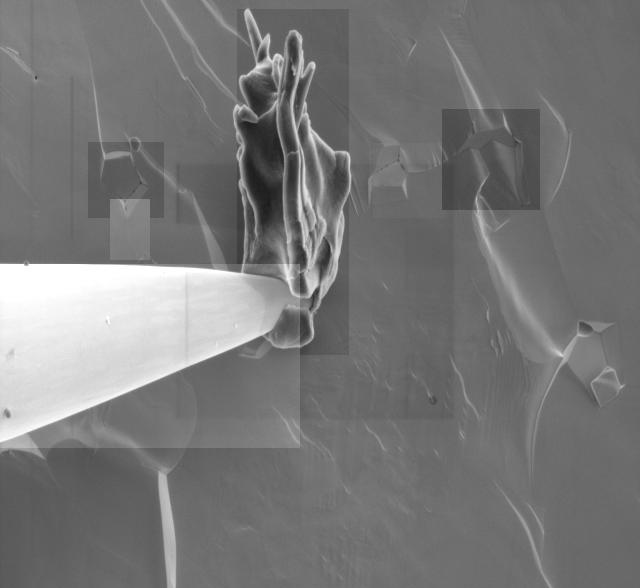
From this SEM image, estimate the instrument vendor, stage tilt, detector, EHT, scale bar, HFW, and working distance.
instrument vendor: FEI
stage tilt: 0°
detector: ETD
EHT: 2 kV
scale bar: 5000 nm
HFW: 27.6 µm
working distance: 5.7 mm